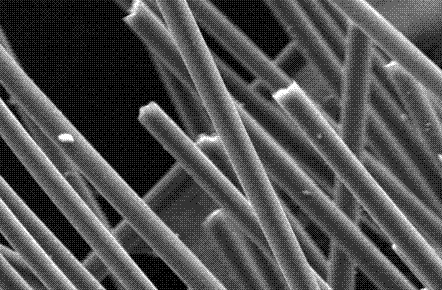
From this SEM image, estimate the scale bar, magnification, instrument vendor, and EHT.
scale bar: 10000 nm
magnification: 1 K X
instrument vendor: JEOL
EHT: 20 kV